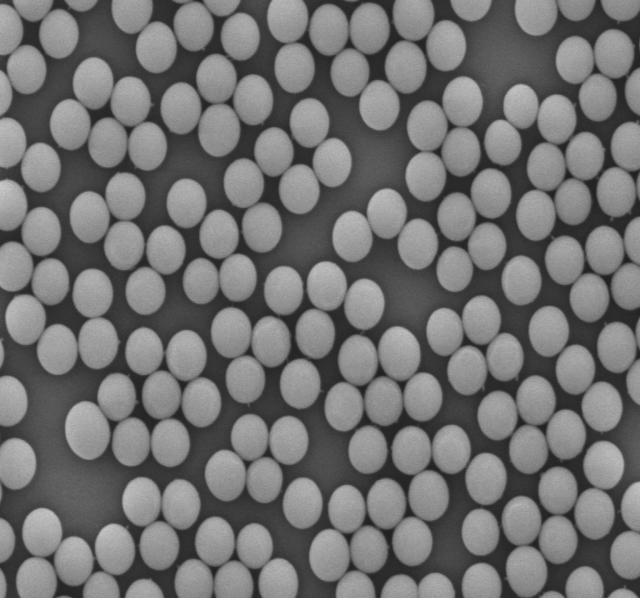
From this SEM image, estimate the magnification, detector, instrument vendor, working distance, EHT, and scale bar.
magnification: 20 K X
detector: LED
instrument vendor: JEOL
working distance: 8 mm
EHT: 5 kV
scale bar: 1000 nm